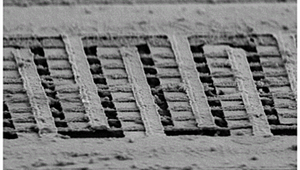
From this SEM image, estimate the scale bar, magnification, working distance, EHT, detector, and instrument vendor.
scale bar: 1000 nm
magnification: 13.54 K X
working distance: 8.1 mm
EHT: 5 kV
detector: SE2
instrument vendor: Zeiss